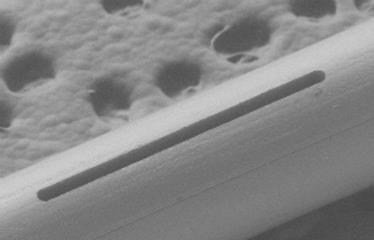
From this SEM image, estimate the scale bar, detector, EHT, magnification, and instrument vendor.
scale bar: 200000 nm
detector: BEI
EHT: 15 kV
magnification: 0.1 K X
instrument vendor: JEOL